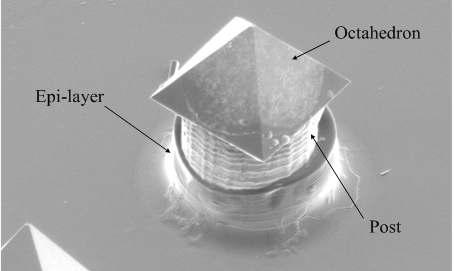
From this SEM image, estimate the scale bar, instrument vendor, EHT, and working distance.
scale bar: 20000 nm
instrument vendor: FEI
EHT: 5 kV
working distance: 25.7 mm